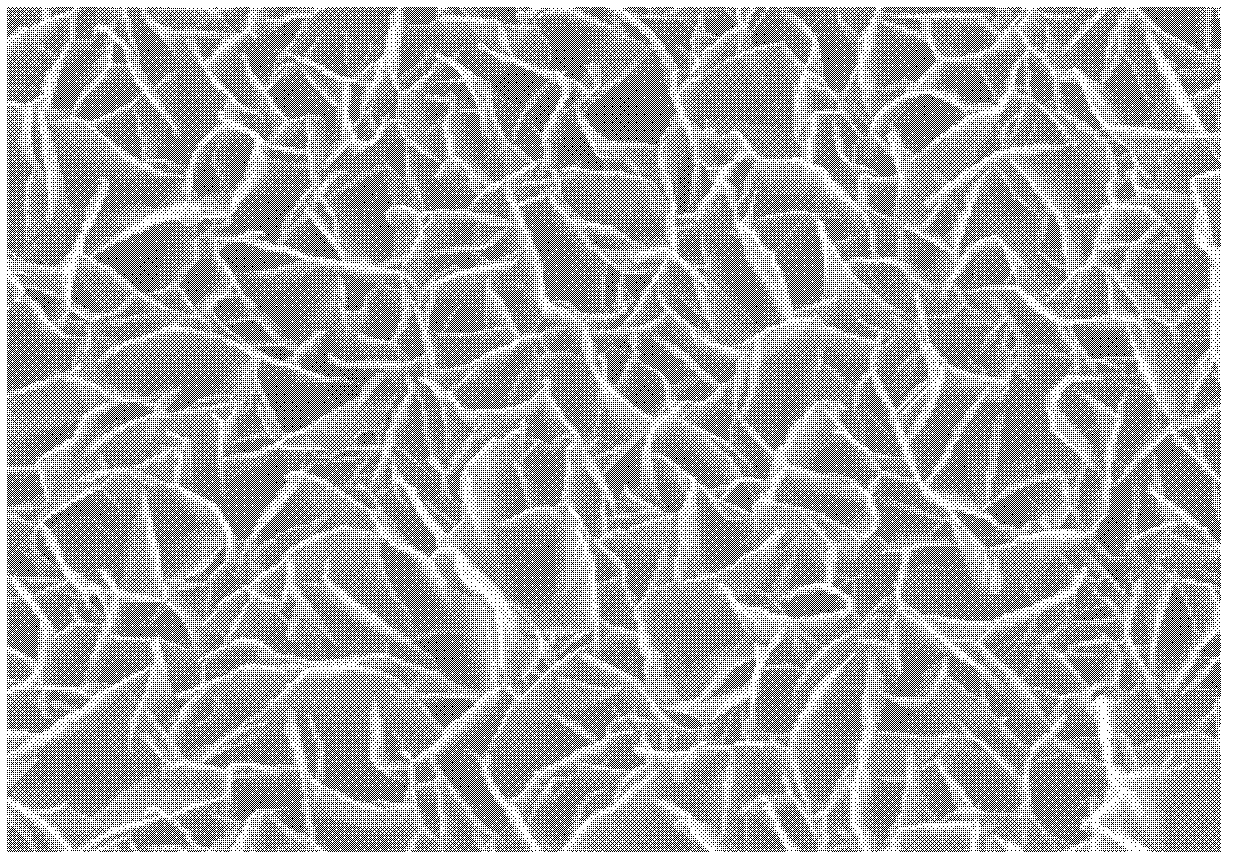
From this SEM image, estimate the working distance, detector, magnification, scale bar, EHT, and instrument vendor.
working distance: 5.4 mm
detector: InLens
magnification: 20 K X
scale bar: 1000 nm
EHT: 20 kV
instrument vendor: Zeiss